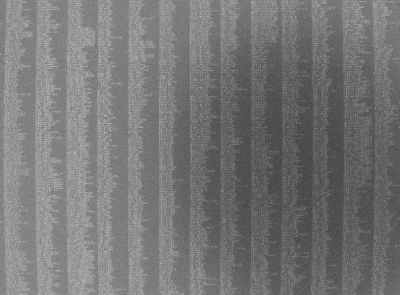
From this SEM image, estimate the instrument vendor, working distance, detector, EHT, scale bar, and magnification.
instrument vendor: JEOL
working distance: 7.5 mm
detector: SEI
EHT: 20 kV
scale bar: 10000 nm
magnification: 0.5 K X